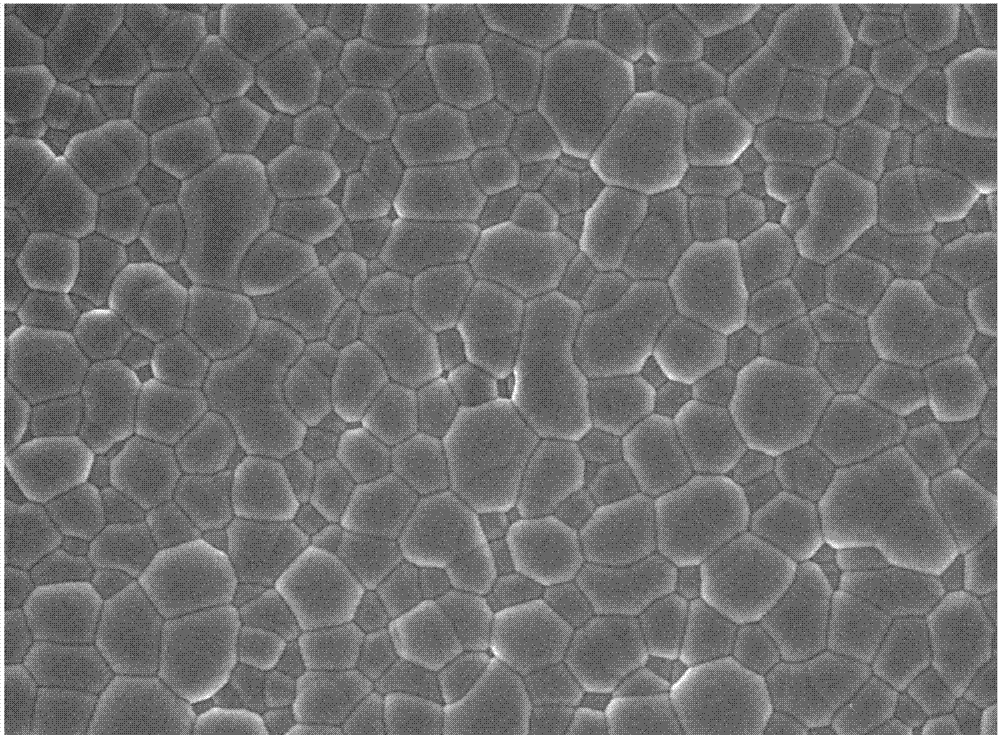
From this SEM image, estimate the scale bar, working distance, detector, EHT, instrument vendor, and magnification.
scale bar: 10000 nm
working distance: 6.7 mm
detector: SEI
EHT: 10 kV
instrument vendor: JEOL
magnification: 2.7 K X